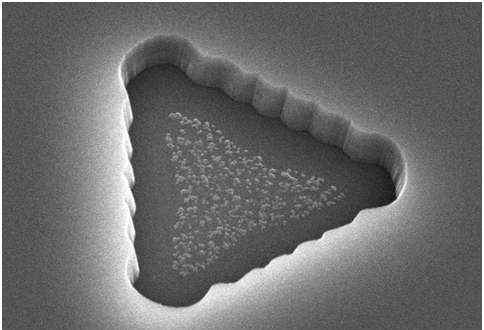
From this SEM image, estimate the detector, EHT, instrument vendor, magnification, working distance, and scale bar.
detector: SE(M)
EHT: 15 kV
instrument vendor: Hitachi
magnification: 13 K X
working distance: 10.4 mm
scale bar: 4000 nm